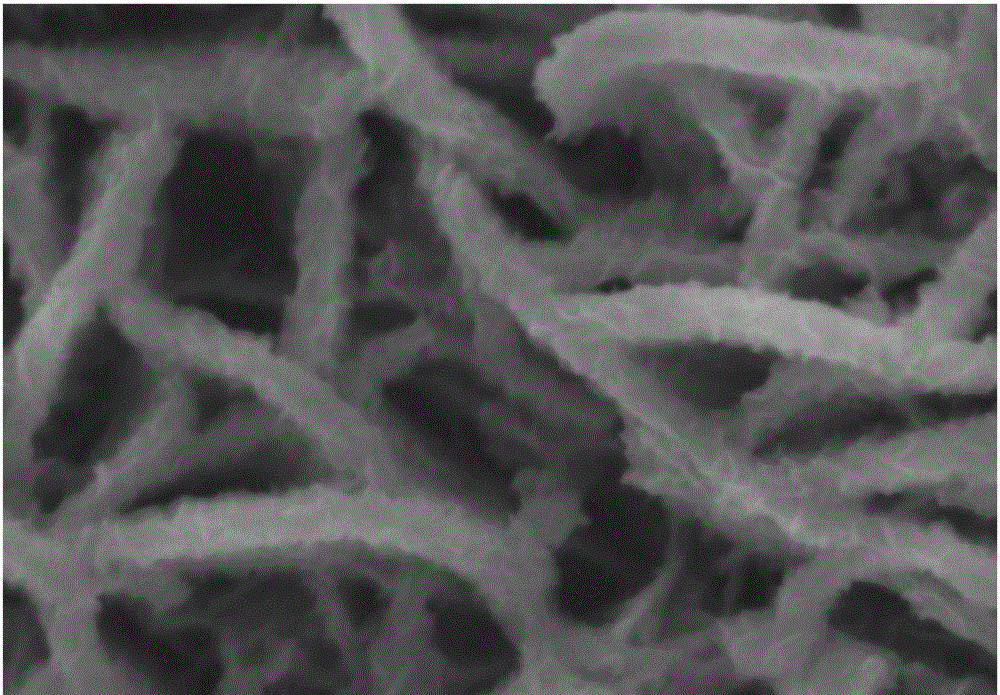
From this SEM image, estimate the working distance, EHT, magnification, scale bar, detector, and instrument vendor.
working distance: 4 mm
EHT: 3 kV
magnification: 100 K X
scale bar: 100 nm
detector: UED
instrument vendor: JEOL